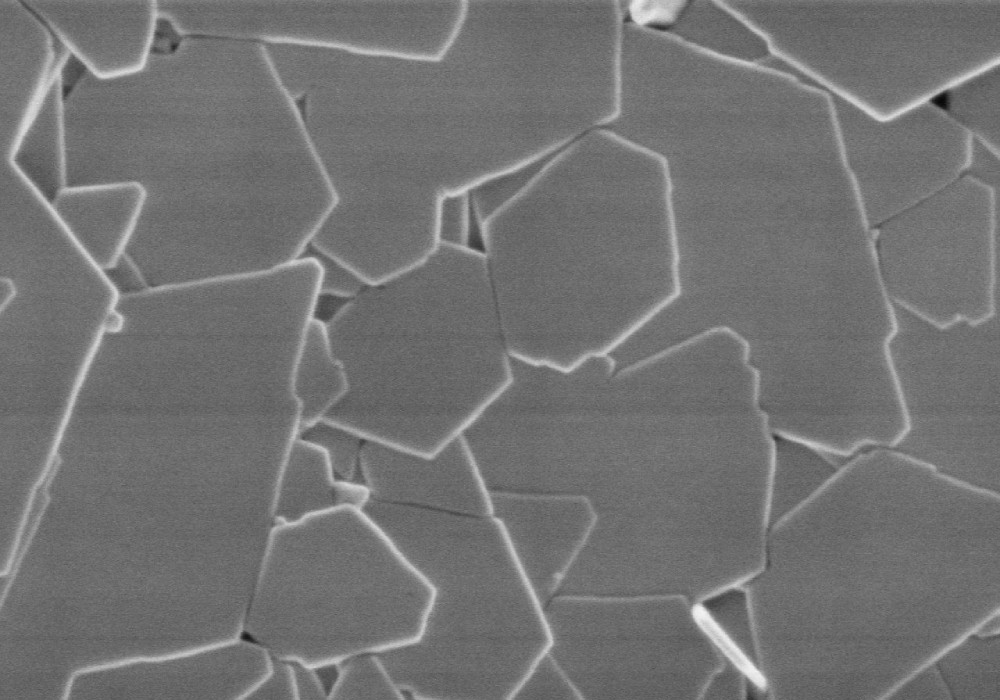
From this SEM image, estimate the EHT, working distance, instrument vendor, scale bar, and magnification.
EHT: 1 kV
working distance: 9.1 mm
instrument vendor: Hitachi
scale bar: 1000 nm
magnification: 50 K X